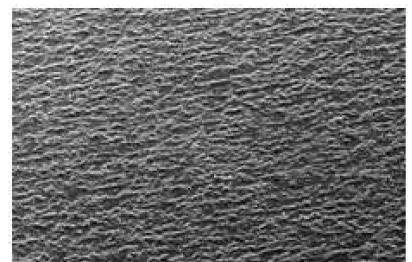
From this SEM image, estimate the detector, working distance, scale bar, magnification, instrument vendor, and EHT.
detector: SE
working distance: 15.1 mm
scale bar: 1000000 nm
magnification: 0.05 K X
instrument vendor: Hitachi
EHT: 25 kV